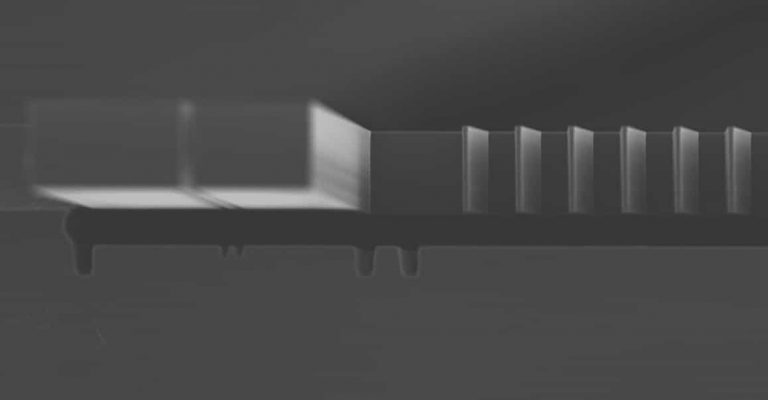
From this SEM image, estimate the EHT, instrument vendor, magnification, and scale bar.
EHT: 16 kV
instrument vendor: JEOL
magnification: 0.2 K X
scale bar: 100000 nm